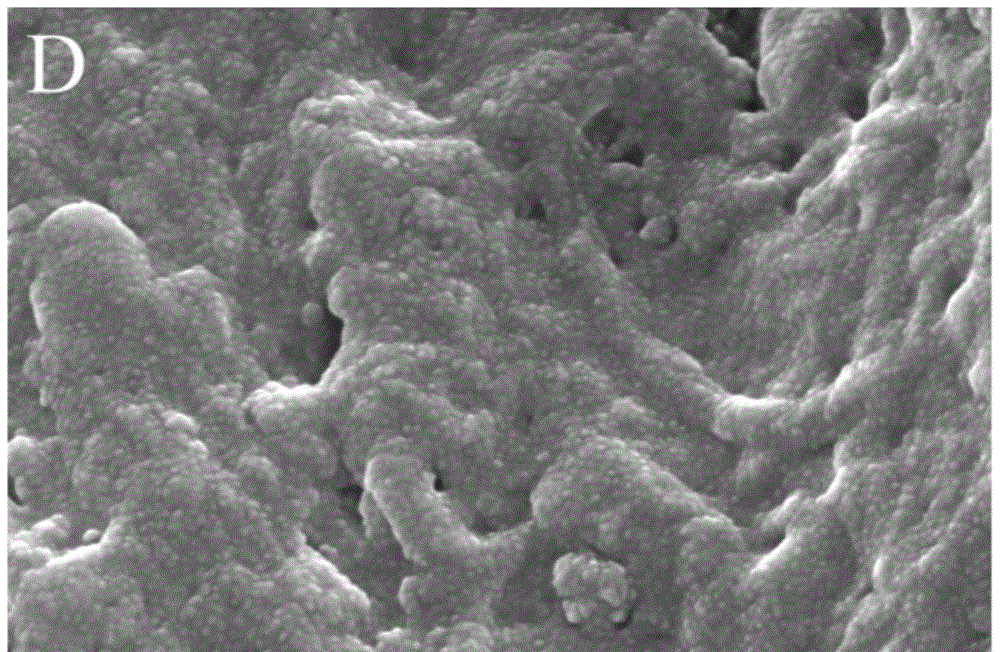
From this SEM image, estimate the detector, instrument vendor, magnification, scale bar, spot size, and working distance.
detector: ETD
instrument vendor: Thermo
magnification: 200 K X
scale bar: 500 nm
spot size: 2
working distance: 9.3 mm